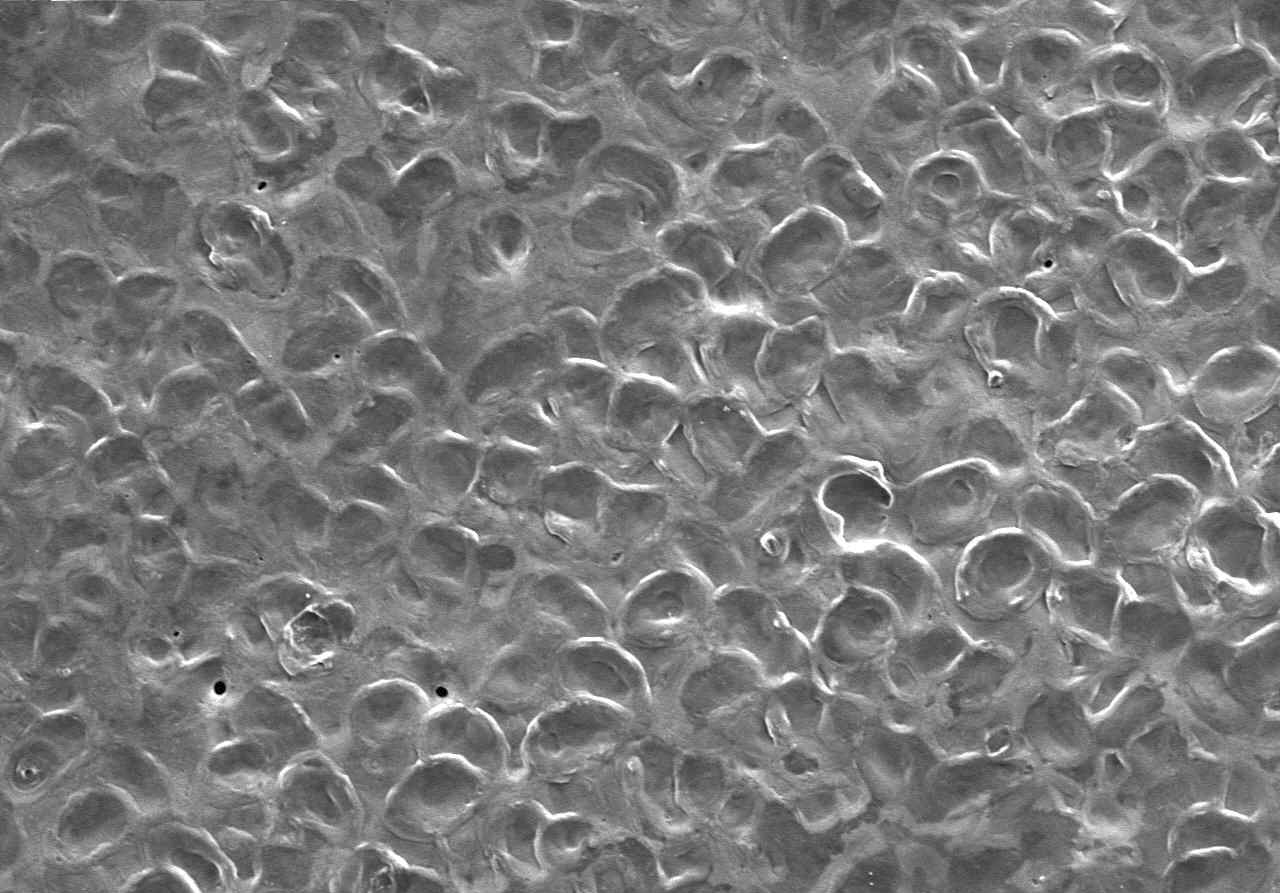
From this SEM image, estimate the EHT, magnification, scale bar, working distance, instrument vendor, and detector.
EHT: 15 kV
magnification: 0.2 K X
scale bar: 200000 nm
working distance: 13.8 mm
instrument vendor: Hitachi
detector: SE(U)